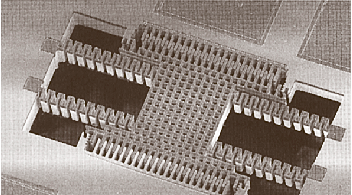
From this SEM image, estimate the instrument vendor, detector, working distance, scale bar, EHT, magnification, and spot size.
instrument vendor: FEI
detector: SE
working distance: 13 mm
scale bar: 100000 nm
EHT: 2 kV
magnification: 0.3 K X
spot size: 5